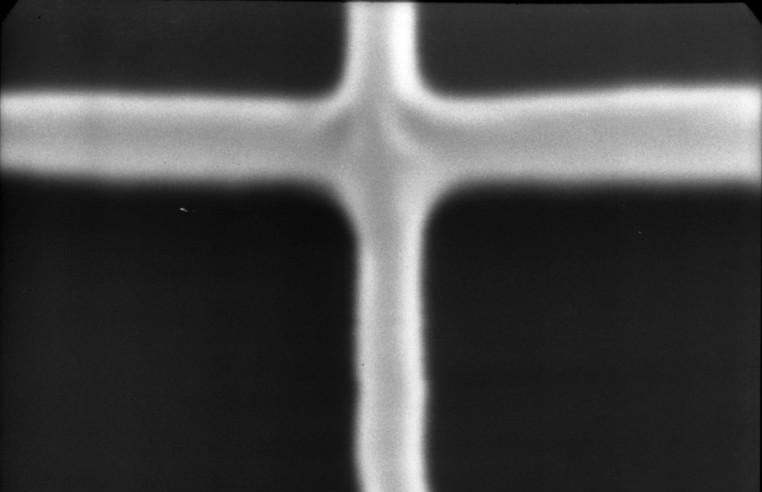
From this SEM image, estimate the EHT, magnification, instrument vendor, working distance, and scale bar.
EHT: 20 kV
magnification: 20 K X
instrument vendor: JEOL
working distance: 48 mm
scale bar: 1000 nm